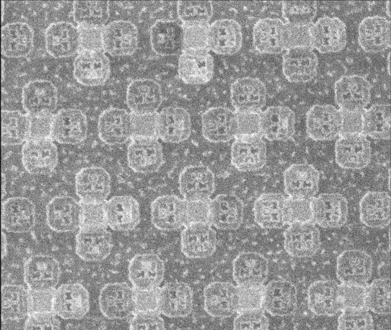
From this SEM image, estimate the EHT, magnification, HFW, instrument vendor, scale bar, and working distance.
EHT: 30 kV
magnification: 40 K X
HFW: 6.78 µm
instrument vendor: FEI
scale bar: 2000 nm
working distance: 30.3 mm